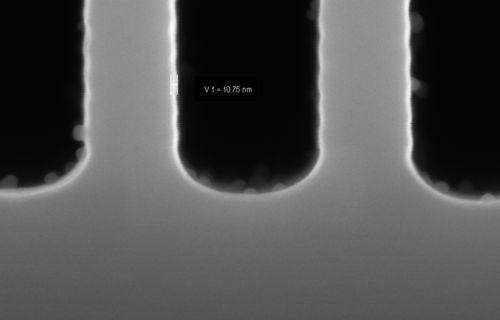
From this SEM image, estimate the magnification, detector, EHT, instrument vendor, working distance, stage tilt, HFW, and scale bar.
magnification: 325.81 K X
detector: InLens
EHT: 10 kV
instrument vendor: Zeiss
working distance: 3.2 mm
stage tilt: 0°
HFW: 0.8471 µm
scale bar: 100 nm